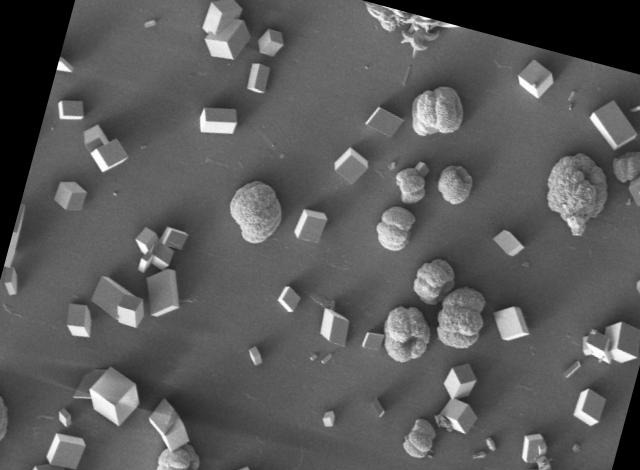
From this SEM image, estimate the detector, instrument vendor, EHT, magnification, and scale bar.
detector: SE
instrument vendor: Tescan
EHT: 5 kV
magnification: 0.4 K X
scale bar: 200000 nm